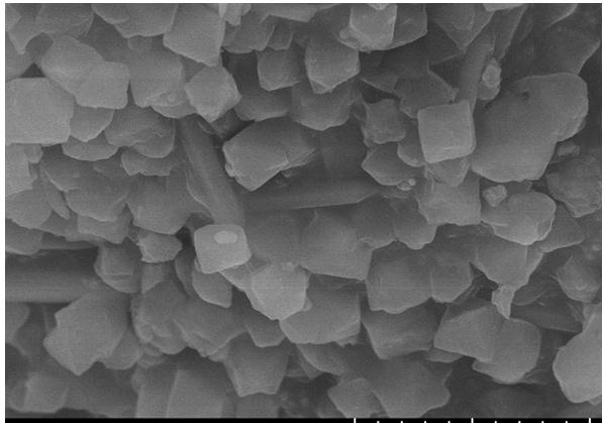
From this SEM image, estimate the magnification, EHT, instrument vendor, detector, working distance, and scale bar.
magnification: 10 K X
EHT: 10 kV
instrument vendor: Hitachi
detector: SE(U)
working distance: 8.7 mm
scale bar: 5000 nm